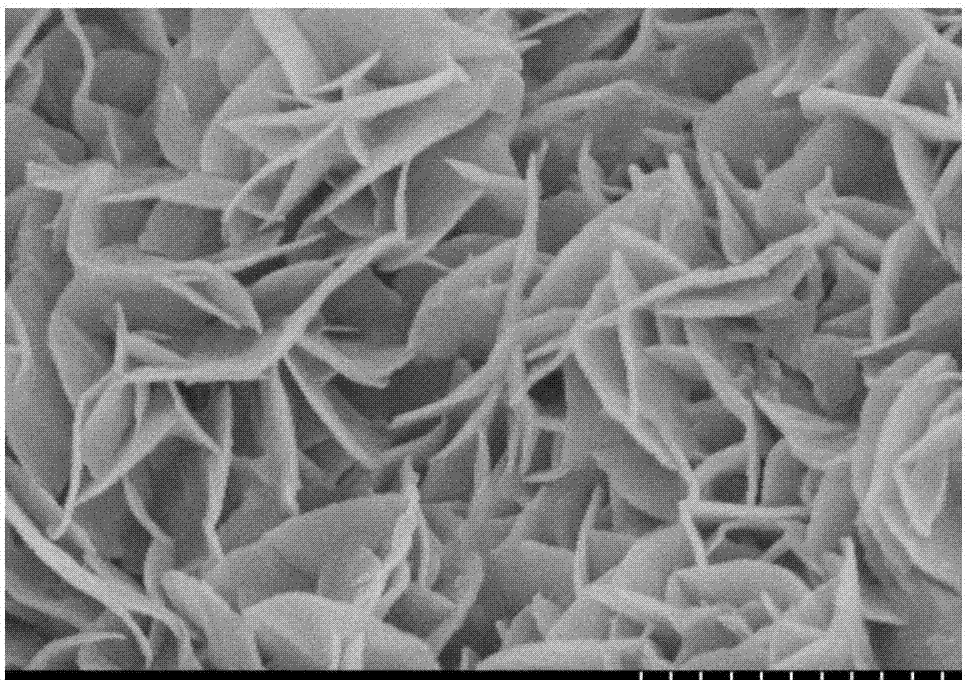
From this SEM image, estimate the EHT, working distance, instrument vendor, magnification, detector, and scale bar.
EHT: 3 kV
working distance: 7.9 mm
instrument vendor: Hitachi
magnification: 40 K X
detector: SE(M)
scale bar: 1000 nm